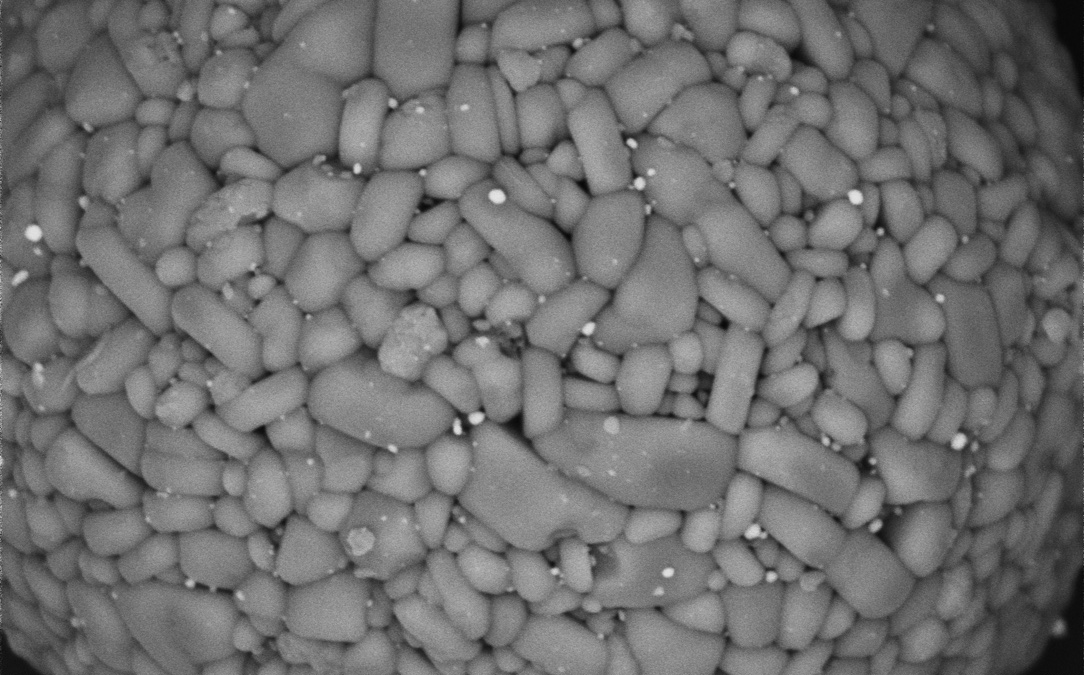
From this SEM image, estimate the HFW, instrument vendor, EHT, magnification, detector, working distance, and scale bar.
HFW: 12.7 µm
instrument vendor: Phenom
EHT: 10 kV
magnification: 10 K X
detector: BSD Full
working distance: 6.739 mm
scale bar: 3000 nm